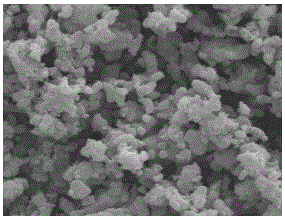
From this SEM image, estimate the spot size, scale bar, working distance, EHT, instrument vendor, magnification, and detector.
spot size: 3.5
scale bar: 2000 nm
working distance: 11.4 mm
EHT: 15 kV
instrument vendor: FEI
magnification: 10 K X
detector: ETD